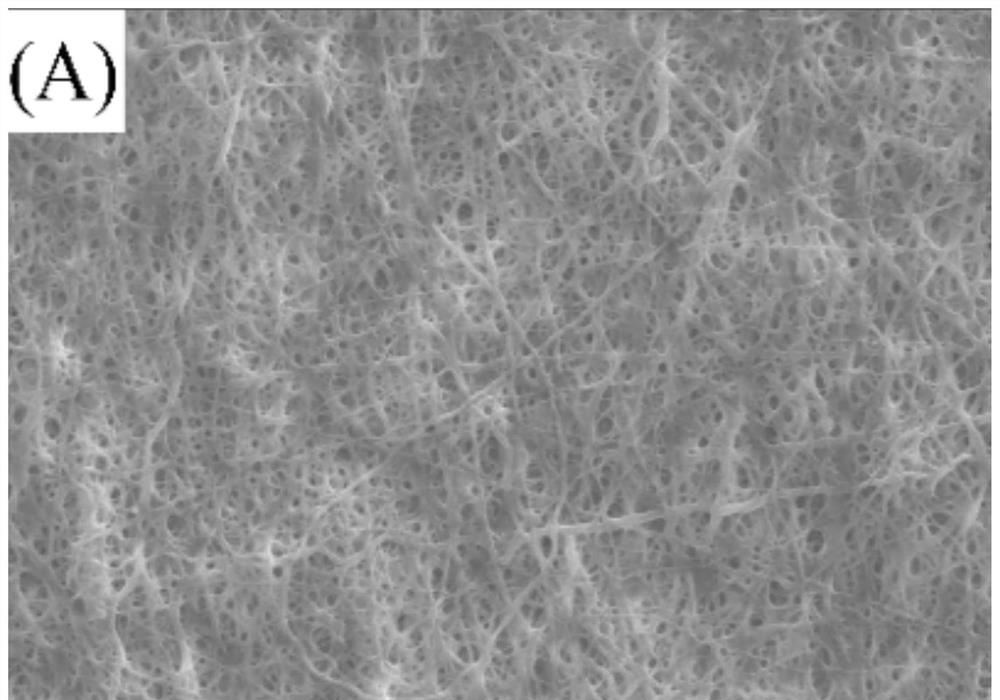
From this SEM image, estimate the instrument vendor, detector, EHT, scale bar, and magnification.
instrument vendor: JEOL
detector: SEI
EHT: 20 kV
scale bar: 10000 nm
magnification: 1 K X